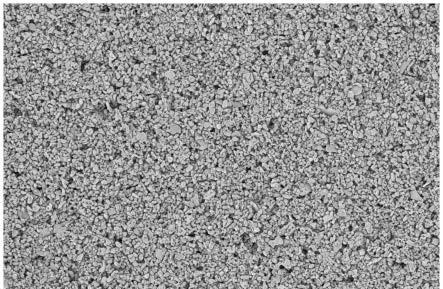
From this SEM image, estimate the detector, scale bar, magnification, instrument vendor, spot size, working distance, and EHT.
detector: T1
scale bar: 10000 nm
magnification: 2 K X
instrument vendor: Thermo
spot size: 9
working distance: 2.8013 mm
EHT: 1 kV